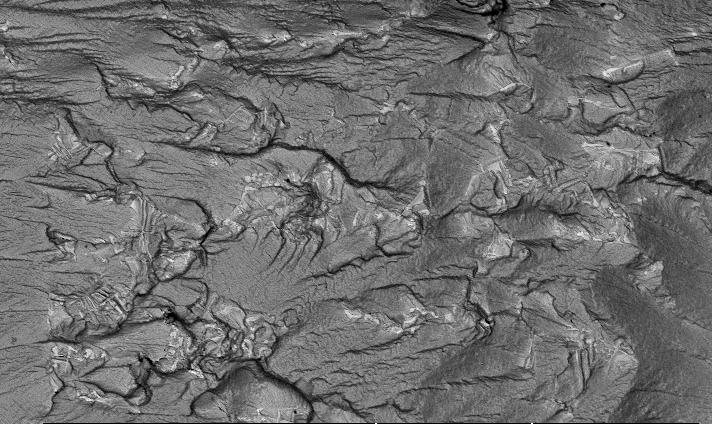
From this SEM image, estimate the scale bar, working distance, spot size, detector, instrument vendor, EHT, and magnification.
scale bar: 200000 nm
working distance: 20.4 mm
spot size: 3.9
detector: BSE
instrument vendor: FEI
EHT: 20 kV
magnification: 0.1 K X